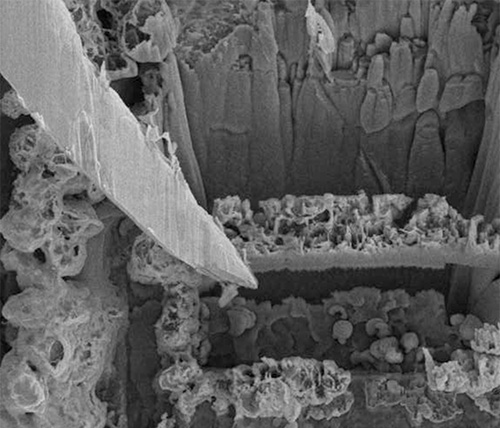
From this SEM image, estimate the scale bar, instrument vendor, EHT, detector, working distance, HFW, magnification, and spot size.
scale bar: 5000 nm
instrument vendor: FEI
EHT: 10 kV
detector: SED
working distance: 4.929 mm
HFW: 46.8 µm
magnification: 6.5 K X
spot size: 3